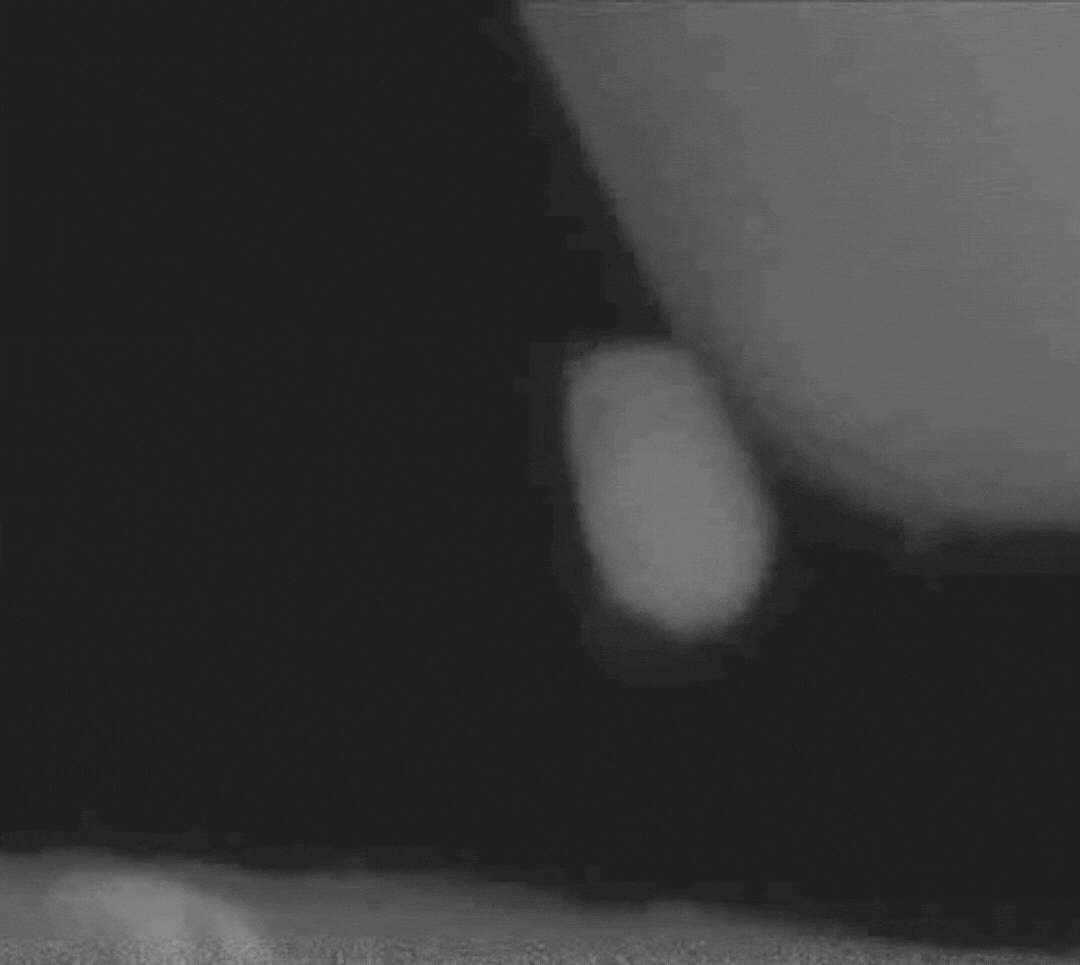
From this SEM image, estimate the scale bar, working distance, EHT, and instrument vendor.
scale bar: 100 nm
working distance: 3 mm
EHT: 5 kV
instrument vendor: Zeiss